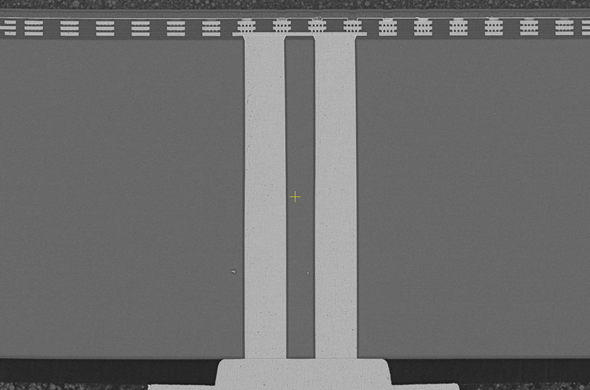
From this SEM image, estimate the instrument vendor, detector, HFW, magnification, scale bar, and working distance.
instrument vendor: Thermo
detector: T1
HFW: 166 µm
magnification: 2.5 K X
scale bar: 50000 nm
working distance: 3.8 mm